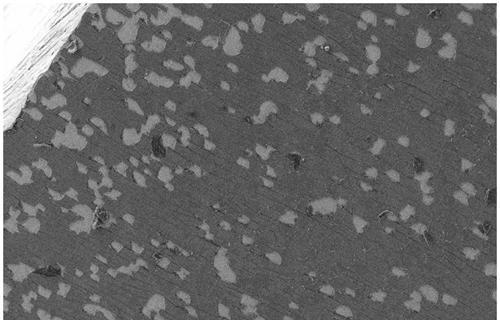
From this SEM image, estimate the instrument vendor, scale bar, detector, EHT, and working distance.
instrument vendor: Zeiss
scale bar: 100000 nm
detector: SE2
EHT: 10 kV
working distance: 3.8 mm